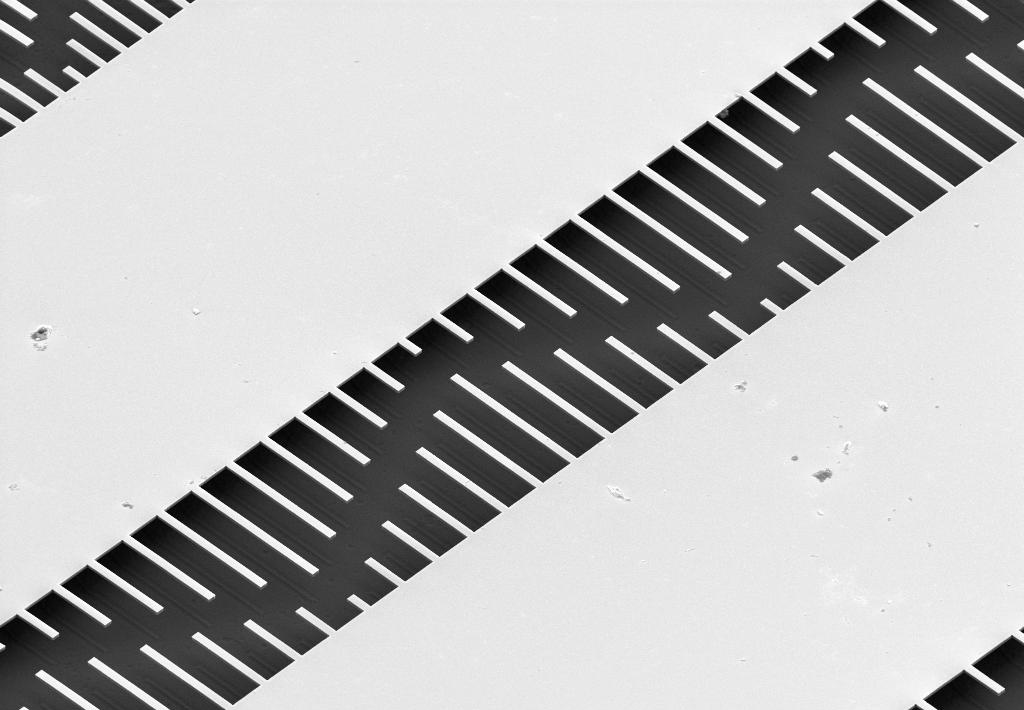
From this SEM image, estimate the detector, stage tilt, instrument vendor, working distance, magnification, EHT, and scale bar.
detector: SE2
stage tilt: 45°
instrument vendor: Zeiss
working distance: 15.3 mm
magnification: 1.1 K X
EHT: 5 kV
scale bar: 2000 nm